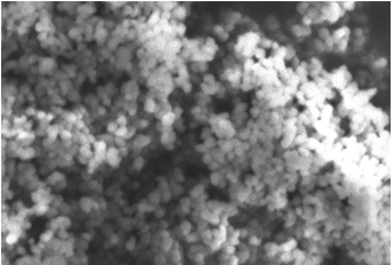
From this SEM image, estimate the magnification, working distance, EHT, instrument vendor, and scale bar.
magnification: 20 K X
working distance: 9 mm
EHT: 5 kV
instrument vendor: other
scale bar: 500 nm